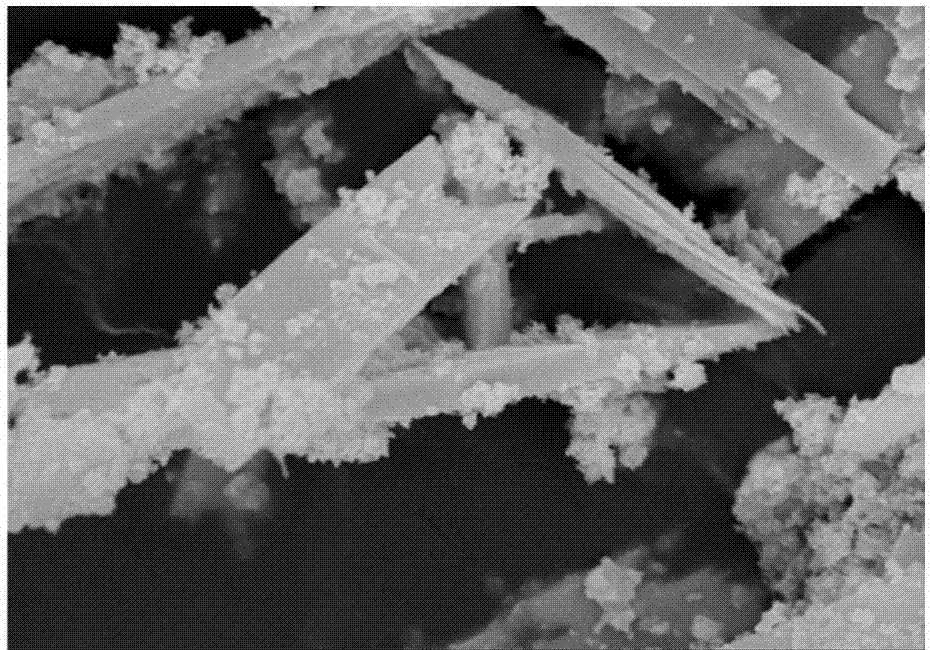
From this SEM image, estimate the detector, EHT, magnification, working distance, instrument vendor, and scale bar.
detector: SE(U)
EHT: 15 kV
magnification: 10 K X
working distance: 6.6 mm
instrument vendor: Hitachi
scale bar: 5000 nm